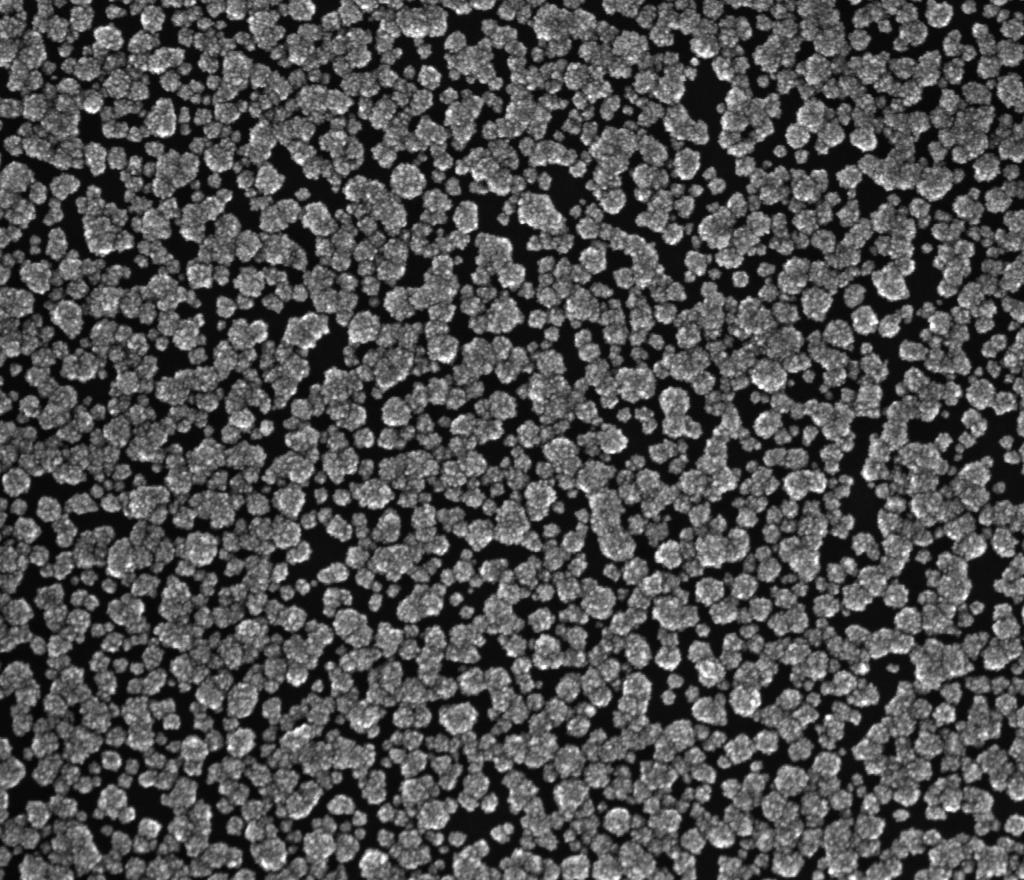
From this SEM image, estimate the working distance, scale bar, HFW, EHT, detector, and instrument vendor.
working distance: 5.8 mm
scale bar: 1000 nm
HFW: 2.56 µm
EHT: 20 kV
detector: TLD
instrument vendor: FEI